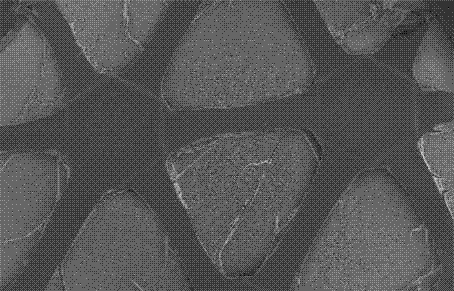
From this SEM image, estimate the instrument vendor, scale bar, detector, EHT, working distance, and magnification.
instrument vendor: Hitachi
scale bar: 1000000 nm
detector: SE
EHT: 15 kV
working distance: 9.6 mm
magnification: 0.035 K X